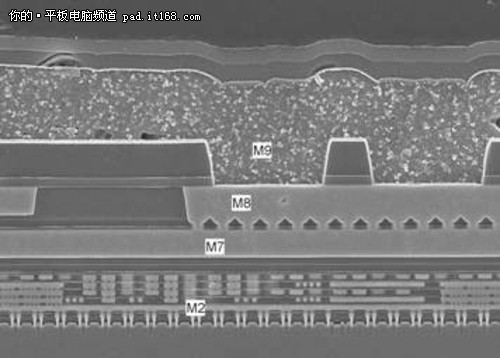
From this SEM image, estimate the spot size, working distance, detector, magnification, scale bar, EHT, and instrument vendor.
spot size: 3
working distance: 5 mm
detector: TLD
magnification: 8 K X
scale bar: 2000 nm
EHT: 10 kV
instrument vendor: FEI